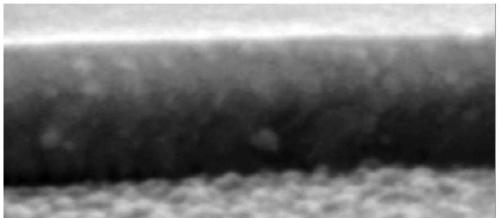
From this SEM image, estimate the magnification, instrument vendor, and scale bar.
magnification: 50 K X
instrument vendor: Zeiss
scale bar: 10 nm